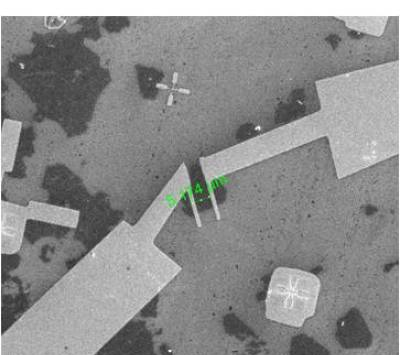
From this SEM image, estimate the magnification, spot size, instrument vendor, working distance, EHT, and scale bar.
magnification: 2.5 K X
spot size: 3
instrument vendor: FEI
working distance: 8.6 mm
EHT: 20 kV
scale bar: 50000 nm